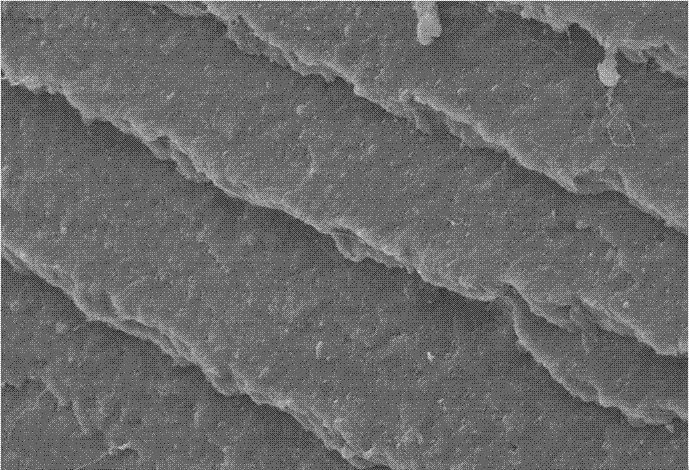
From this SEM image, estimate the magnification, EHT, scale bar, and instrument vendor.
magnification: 0.5 K X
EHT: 20 kV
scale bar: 100000 nm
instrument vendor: other